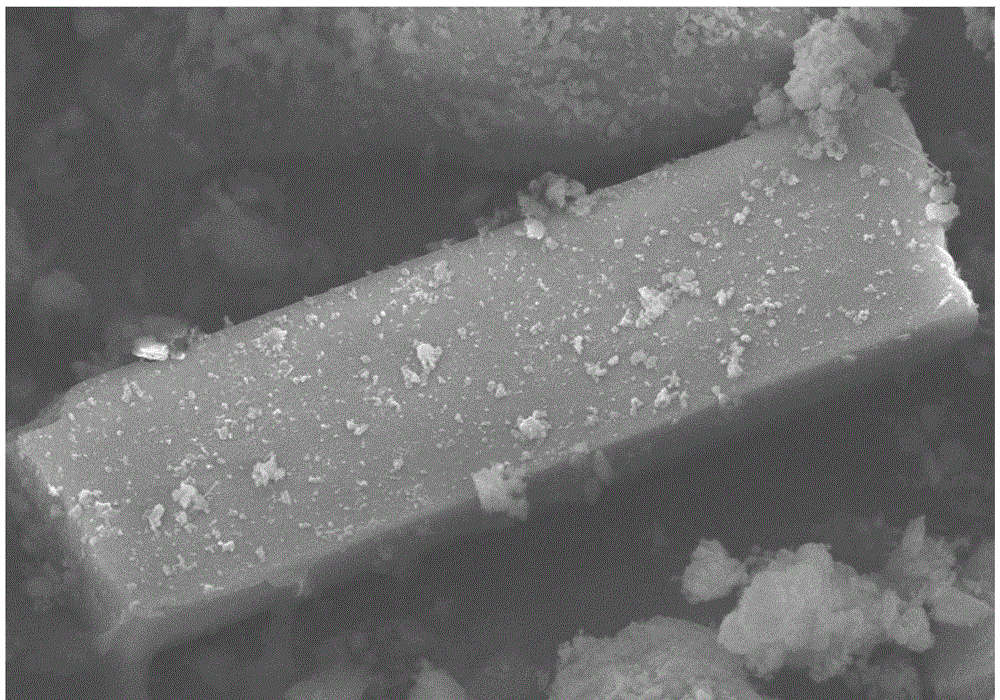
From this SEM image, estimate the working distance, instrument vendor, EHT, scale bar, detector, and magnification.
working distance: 5.9 mm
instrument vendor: Hitachi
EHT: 15 kV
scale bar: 10000 nm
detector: SE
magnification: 3.5 K X